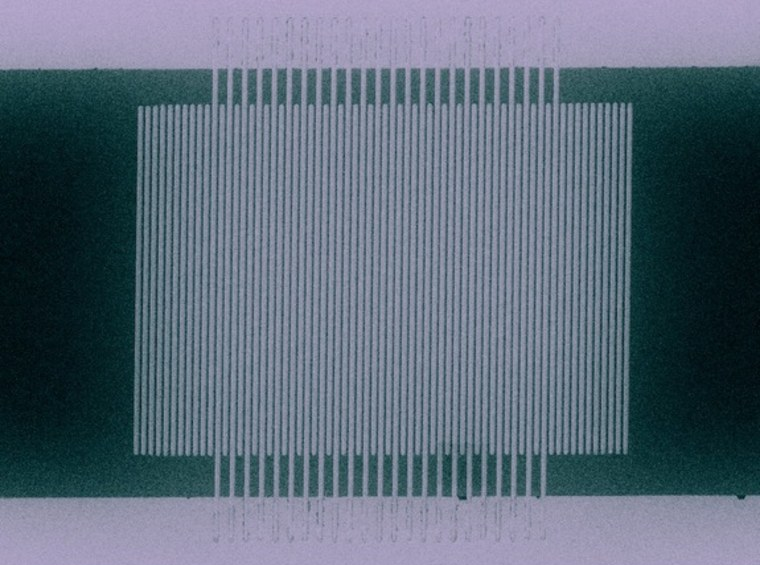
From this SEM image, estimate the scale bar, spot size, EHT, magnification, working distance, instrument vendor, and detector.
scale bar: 5000 nm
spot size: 3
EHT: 30 kV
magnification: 3.5 K X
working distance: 5 mm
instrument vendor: FEI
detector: SE